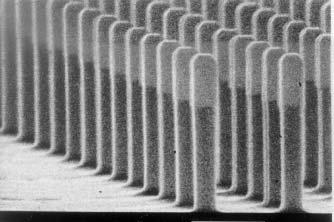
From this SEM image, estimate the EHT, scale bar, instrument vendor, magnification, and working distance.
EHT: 25 kV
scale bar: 1000 nm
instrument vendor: JEOL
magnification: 25 K X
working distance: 44 mm